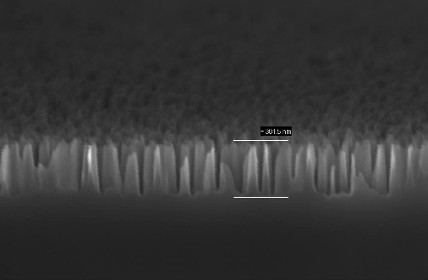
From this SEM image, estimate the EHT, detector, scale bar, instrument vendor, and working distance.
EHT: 5 kV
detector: InLens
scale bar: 200 nm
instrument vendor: Zeiss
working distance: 1 mm